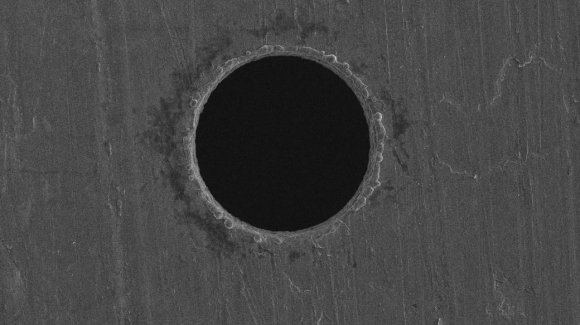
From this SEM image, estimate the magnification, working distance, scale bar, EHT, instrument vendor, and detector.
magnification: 1 K X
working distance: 7.9 mm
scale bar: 50000 nm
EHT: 5 kV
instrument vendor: Hitachi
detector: SE